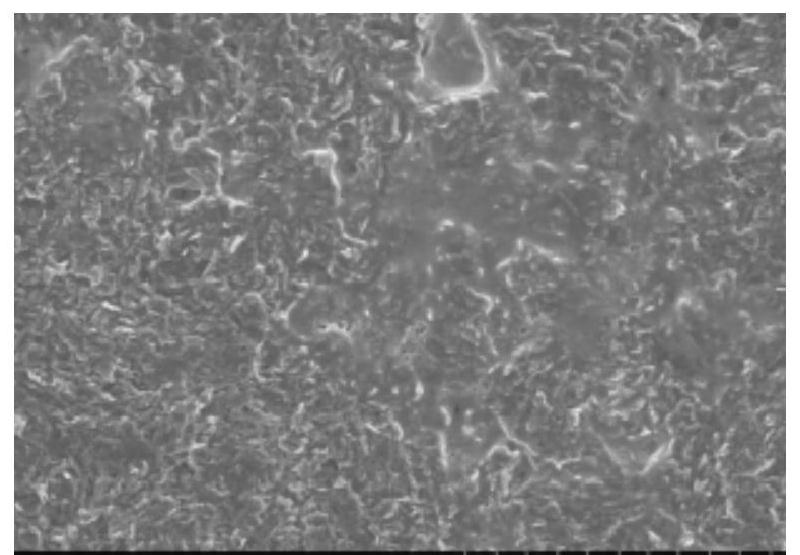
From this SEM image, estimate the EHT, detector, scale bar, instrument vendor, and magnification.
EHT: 15 kV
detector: SE(M)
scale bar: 100000 nm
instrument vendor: Hitachi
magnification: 0.5 K X